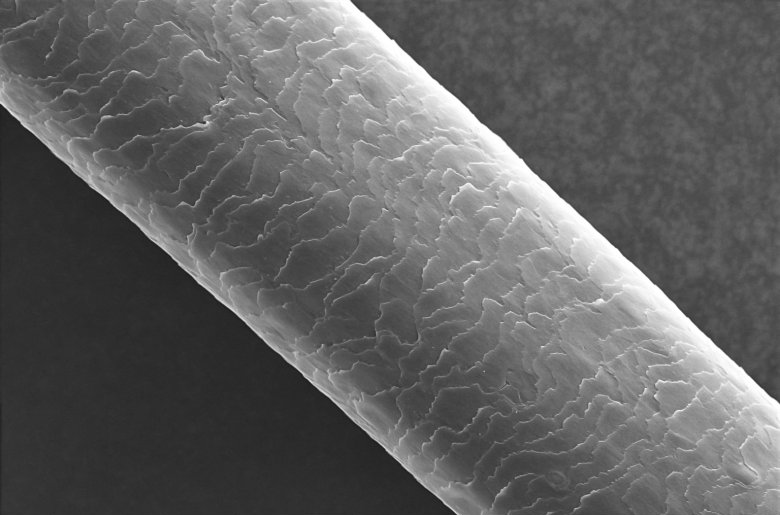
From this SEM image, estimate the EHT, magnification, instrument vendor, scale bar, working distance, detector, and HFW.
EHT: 15 kV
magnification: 0.5 K X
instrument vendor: Thermo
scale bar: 50000 nm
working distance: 8 mm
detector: ETD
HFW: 254 µm